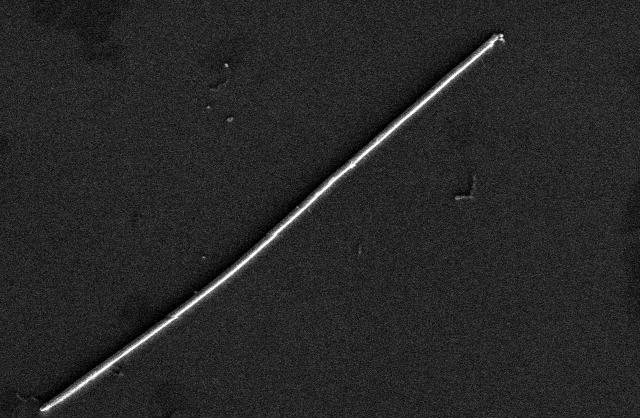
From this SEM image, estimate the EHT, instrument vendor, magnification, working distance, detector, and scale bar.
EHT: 2 kV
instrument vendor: Hitachi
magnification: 8 K X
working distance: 8.6 mm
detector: SE(L)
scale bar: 5000 nm